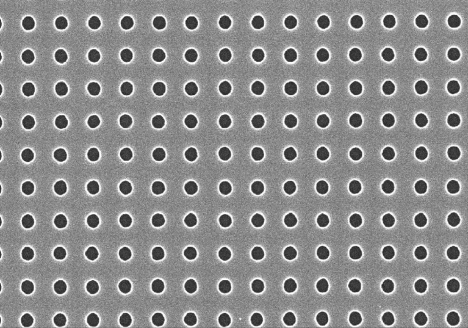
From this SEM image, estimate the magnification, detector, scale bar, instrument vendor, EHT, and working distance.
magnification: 30 K X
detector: SE(U)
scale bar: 1000 nm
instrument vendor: Hitachi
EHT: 10 kV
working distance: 5.1 mm